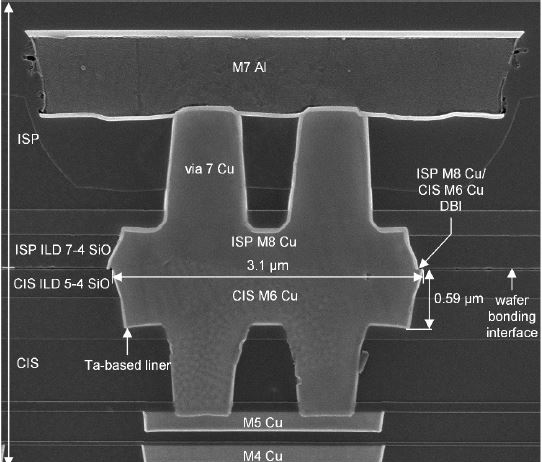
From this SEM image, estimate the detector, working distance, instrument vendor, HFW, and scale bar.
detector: TLD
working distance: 4.9 mm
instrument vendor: FEI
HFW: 5.29 µm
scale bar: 2000 nm